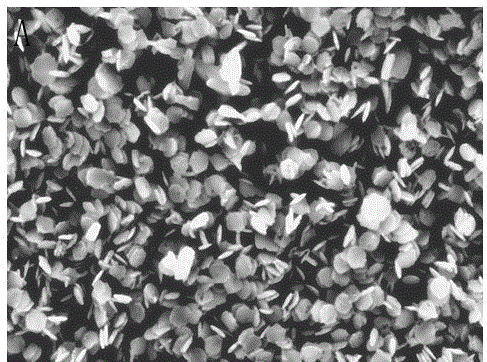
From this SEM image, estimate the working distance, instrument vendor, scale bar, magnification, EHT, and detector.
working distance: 15.7 mm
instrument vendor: Hitachi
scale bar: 5000 nm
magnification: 7.5 K X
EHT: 15 kV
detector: SE